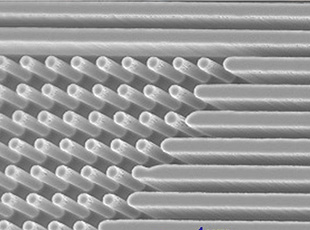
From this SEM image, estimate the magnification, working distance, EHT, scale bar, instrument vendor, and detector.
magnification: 5 K X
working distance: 16 mm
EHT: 5 kV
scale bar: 1000 nm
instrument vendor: JEOL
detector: SEMIMG